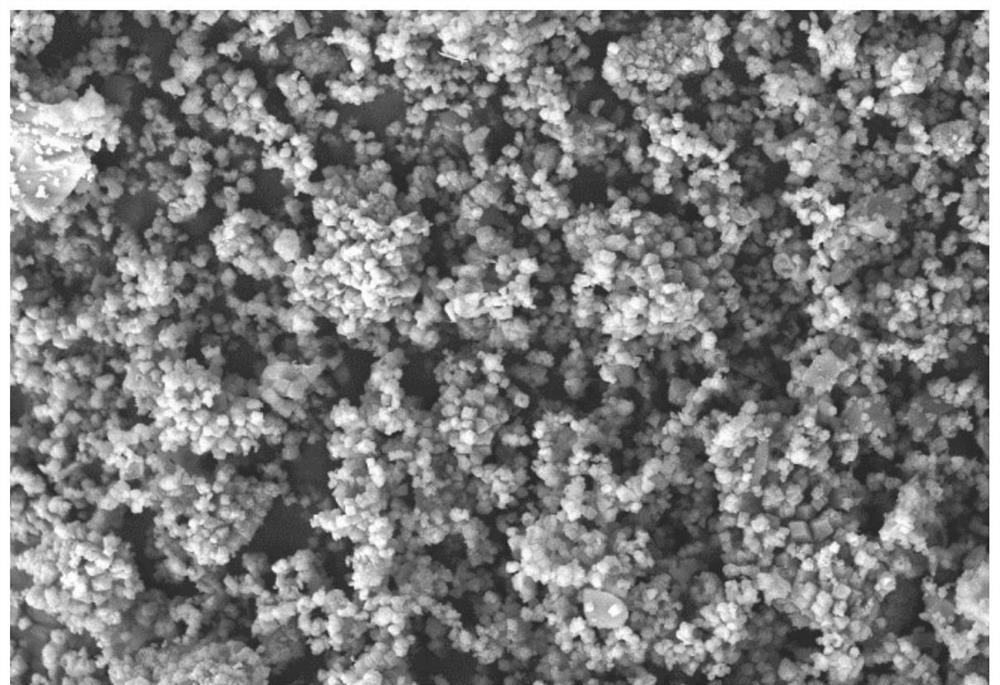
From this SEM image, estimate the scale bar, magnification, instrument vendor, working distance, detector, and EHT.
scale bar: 2000 nm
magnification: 5 K X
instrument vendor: Zeiss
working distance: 7.8 mm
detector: SE2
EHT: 10 kV